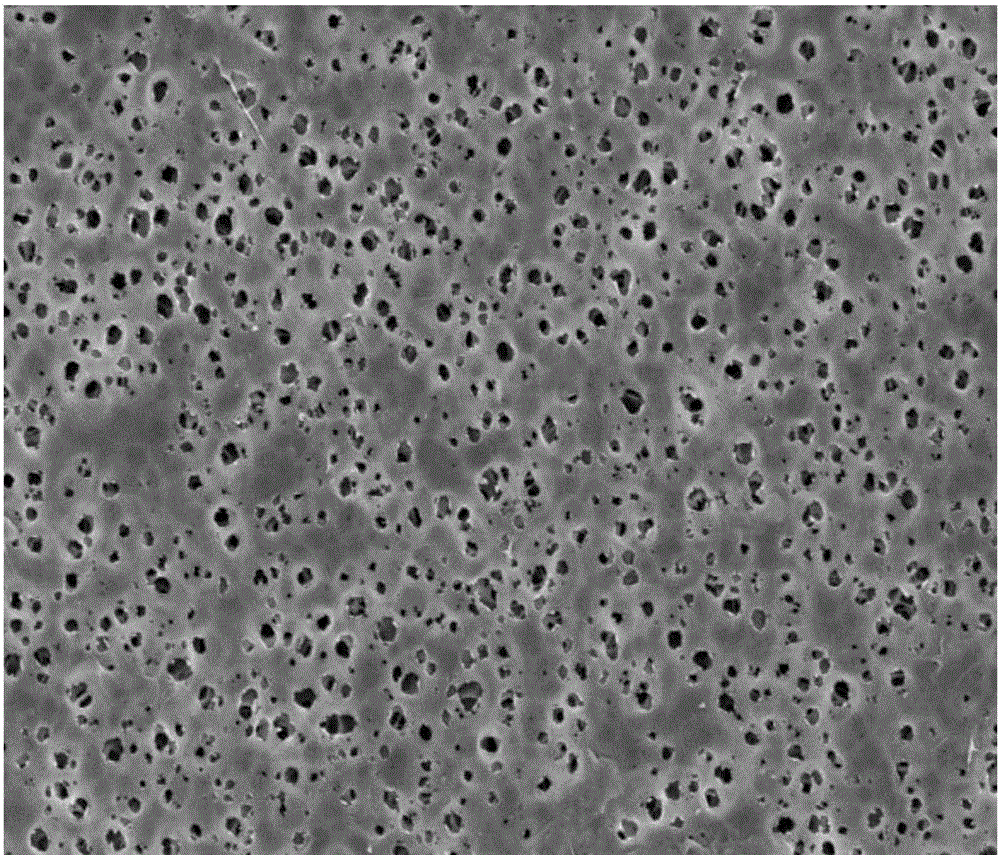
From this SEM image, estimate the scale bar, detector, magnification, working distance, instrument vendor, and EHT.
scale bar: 10000 nm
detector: SE(M)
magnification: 3.5 K X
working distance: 8.7 mm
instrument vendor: Hitachi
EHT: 5 kV